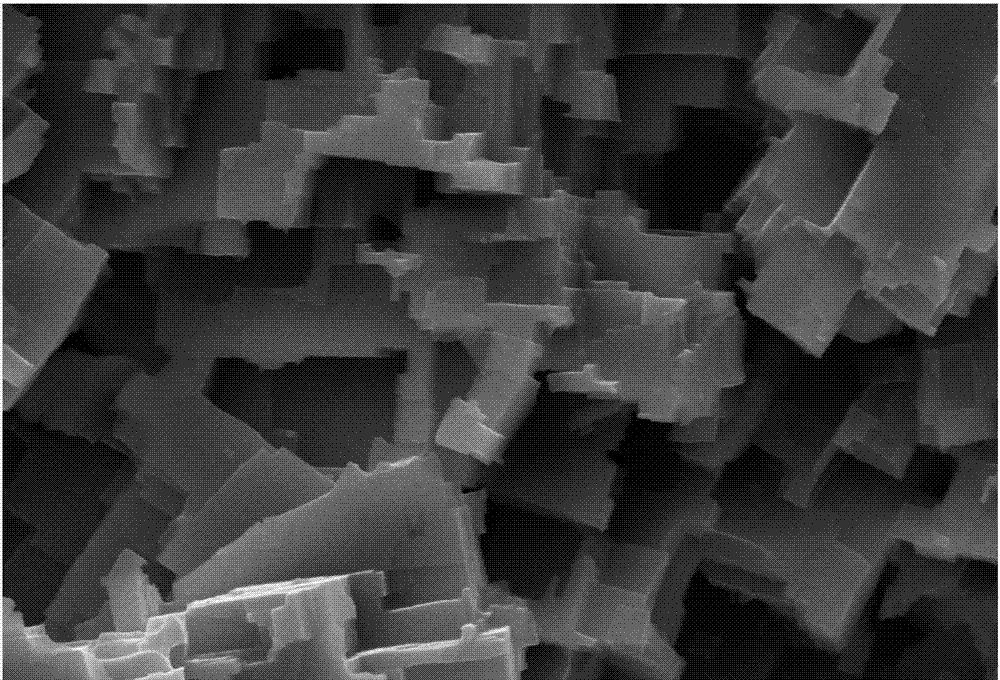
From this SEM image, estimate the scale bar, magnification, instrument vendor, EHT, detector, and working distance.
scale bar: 1000 nm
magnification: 5 K X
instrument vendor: Zeiss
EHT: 15 kV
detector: SE2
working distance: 24.9 mm